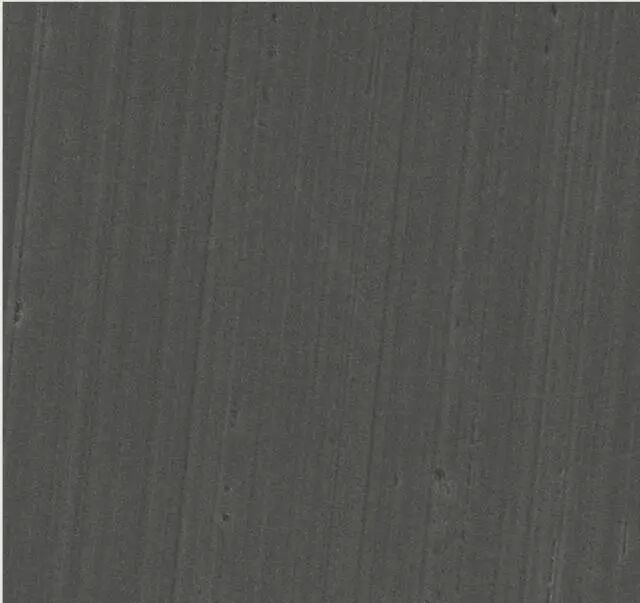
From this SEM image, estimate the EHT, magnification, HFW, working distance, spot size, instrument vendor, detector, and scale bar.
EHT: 20 kV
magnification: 1 K X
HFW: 140 µm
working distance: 14.4 mm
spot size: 5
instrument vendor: FEI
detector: ETD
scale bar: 20000 nm